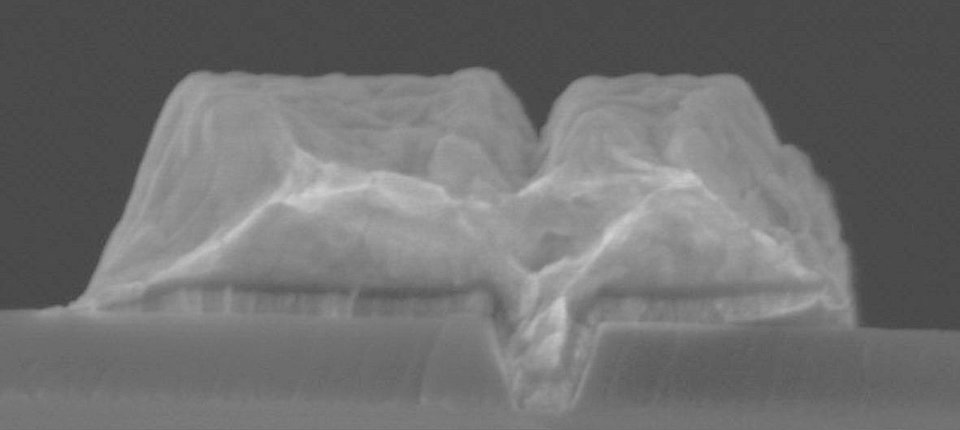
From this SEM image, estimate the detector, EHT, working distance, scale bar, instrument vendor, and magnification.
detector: SE(U)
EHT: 10 kV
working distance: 9.6 mm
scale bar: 500 nm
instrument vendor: Hitachi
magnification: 110 K X